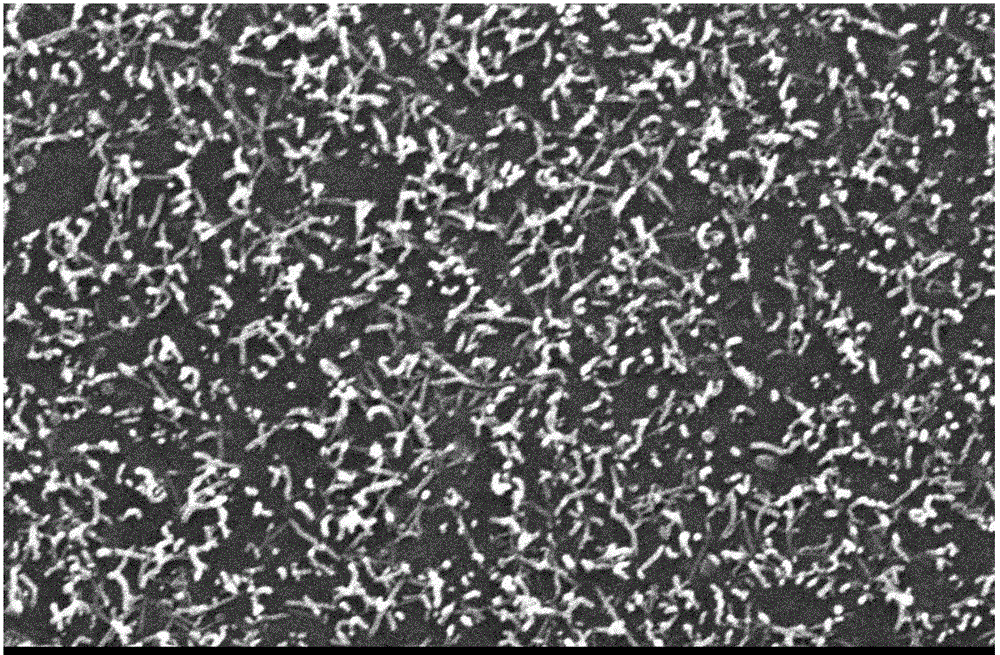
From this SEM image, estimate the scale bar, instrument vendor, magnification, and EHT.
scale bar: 1000 nm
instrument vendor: JEOL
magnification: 10 K X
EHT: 15 kV